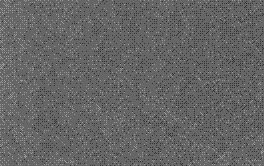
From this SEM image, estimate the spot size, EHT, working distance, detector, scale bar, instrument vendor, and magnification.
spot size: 4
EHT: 25 kV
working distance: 6.8 mm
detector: SE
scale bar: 1000 nm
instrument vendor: FEI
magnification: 50 K X